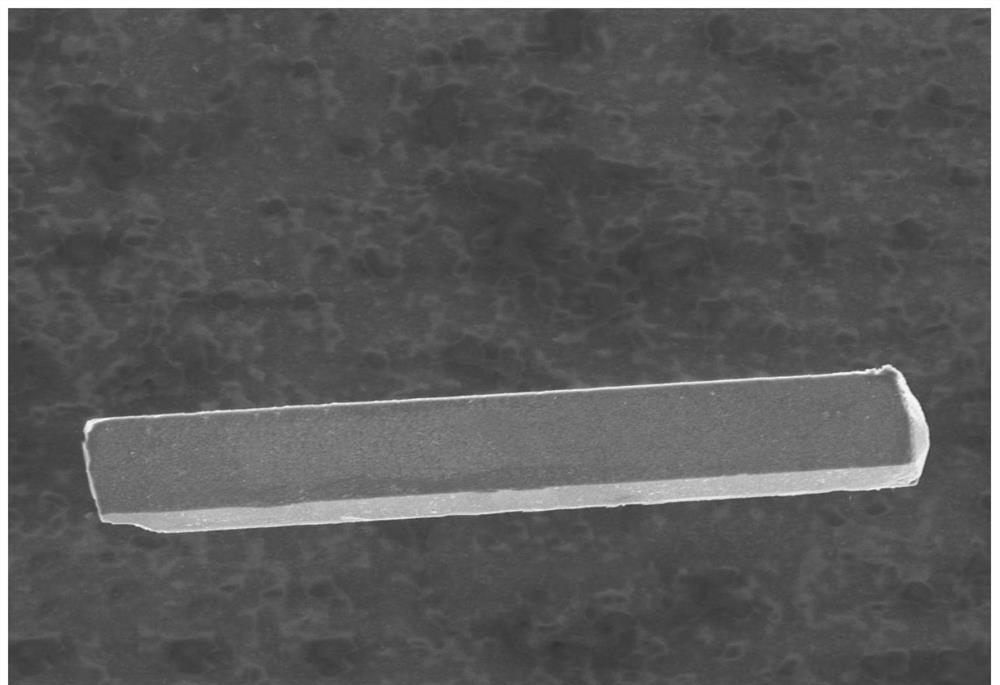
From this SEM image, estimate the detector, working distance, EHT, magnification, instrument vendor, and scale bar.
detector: SE(UL)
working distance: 8.2 mm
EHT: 5 kV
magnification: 1.3 K X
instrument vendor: Hitachi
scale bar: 40000 nm